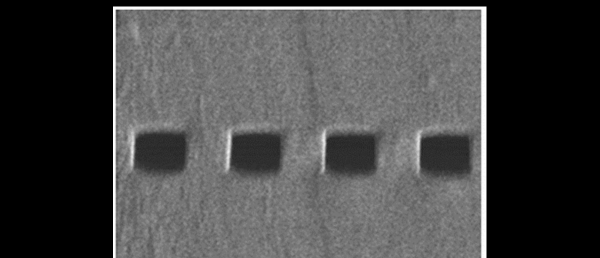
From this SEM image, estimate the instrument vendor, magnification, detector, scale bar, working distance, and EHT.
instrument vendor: Hitachi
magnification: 3.5 K X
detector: SE(L)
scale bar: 10000 nm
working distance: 8.3 mm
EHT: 1 kV